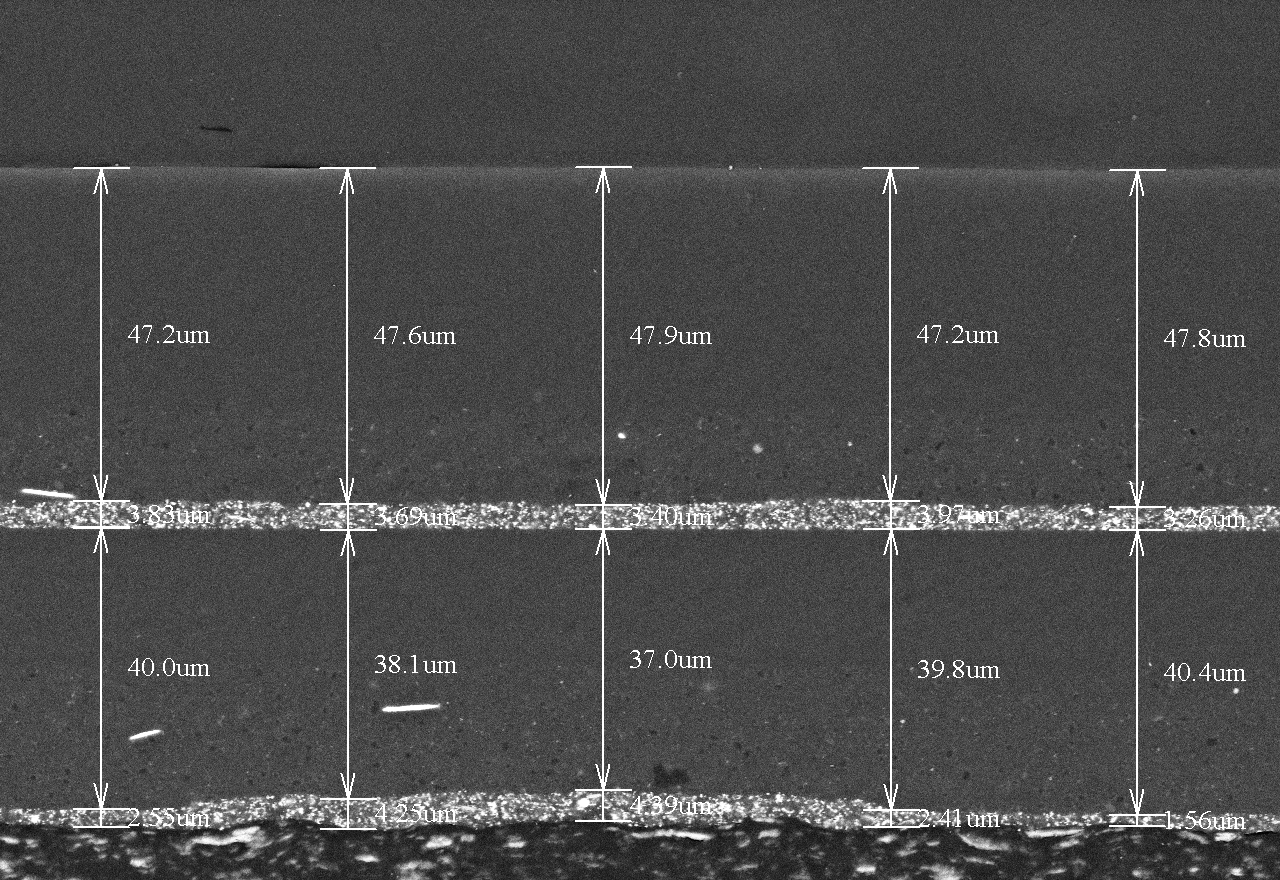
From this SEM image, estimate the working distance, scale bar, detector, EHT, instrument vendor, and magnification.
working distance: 7 mm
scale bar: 50000 nm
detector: BSE3D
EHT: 15 kV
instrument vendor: Hitachi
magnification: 0.7 K X